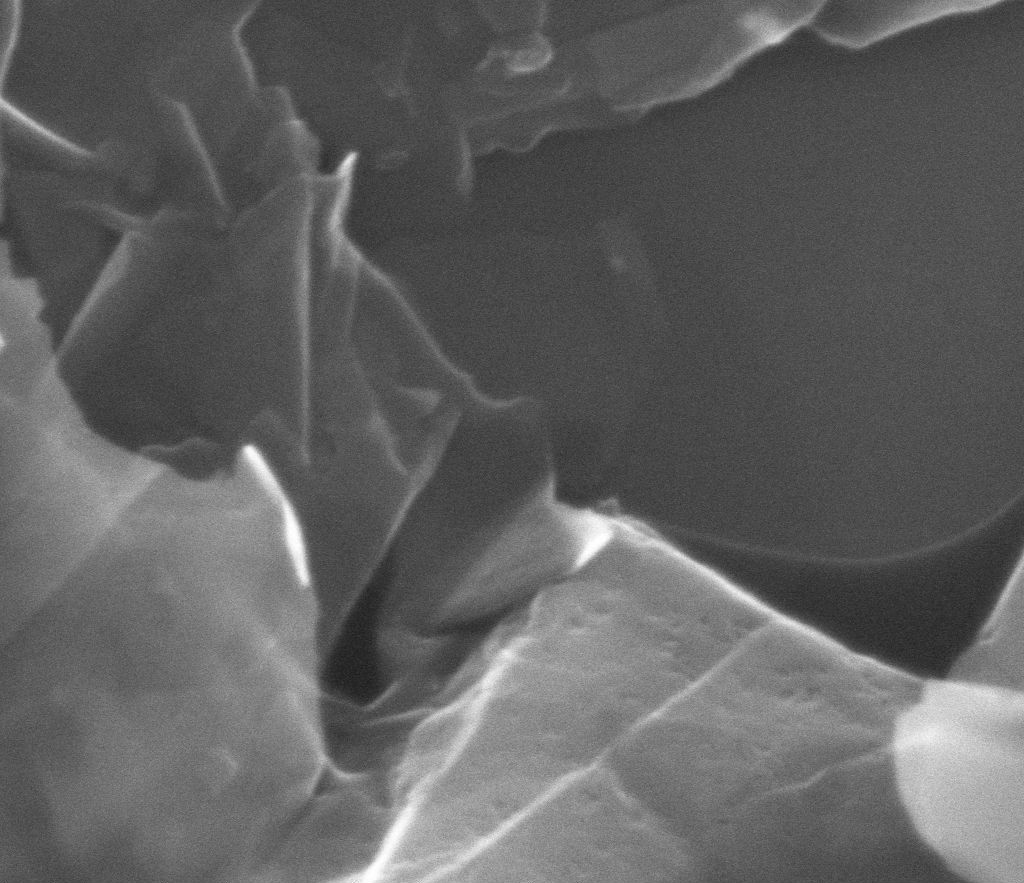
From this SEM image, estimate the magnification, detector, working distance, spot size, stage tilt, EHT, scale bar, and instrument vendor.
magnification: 100 K X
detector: ETD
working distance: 10.4 mm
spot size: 3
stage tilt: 0°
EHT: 10 kV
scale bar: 1000 nm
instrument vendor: FEI